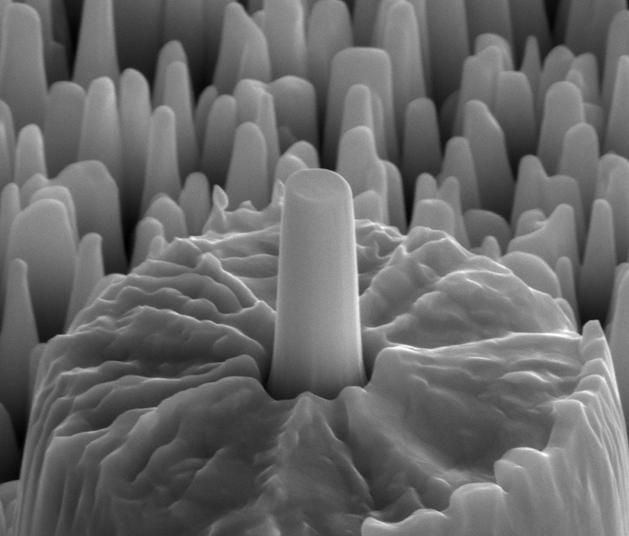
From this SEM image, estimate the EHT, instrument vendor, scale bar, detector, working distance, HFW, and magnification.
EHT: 18 kV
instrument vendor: FEI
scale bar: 2000 nm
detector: ETD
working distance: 5.1 mm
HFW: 5.12 µm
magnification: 50 K X